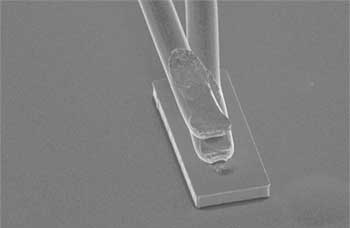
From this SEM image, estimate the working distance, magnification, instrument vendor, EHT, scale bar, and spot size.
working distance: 16.7 mm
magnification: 0.4 K X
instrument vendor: FEI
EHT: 20 kV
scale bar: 50000 nm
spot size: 4